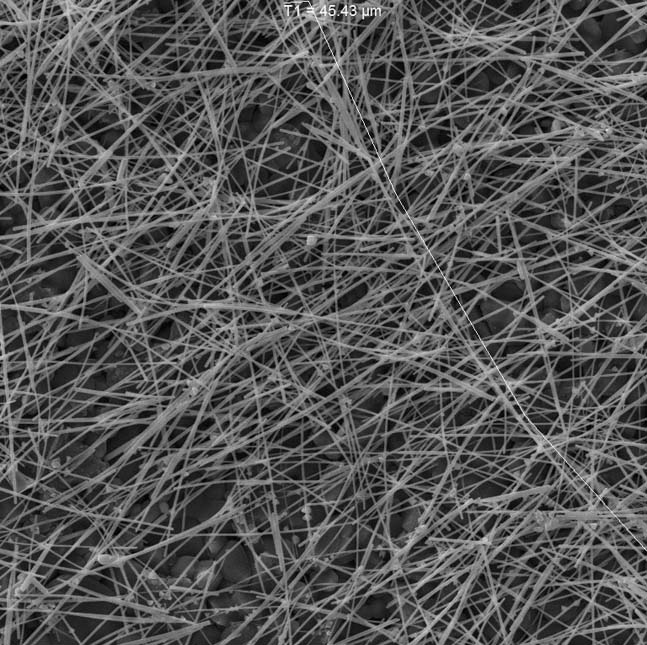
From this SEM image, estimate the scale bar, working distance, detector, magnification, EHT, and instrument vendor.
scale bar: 10000 nm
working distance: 11.71 mm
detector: SE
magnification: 5 K X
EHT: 15 kV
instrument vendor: Tescan